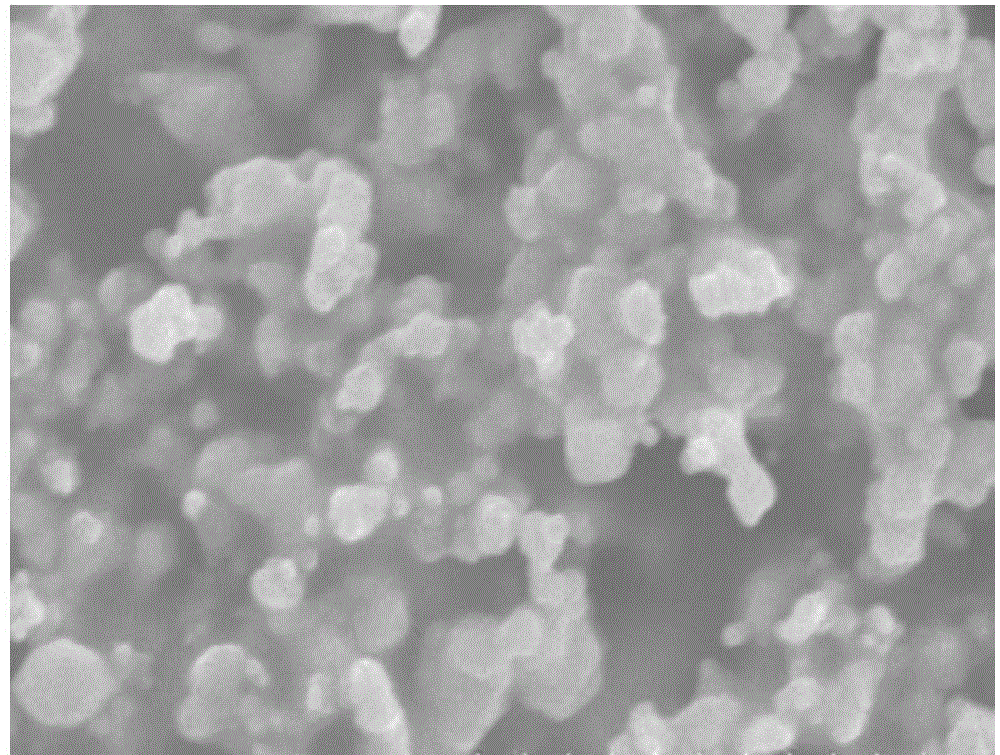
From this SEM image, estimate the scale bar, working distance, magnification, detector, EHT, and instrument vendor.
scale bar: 3000 nm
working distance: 9.6 mm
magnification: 19.1 K X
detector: SE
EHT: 15 kV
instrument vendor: Hitachi